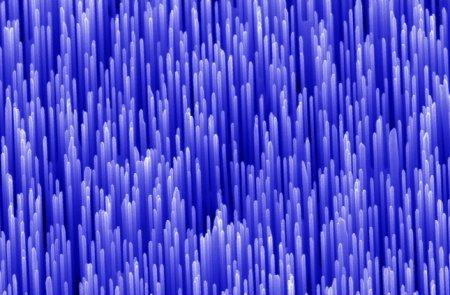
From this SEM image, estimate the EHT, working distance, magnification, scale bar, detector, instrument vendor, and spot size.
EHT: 10 kV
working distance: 13 mm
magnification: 16.215 K X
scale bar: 1000 nm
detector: SE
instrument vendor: FEI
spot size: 3